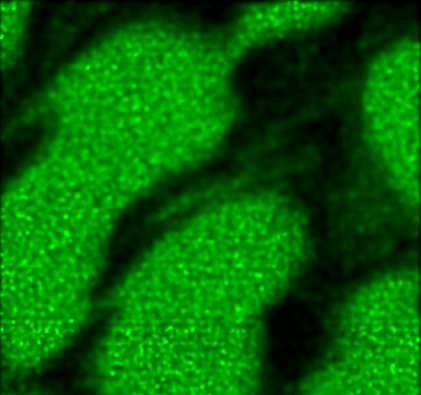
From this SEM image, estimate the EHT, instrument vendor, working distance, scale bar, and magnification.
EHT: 30 kV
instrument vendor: other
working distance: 25.1 mm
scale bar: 20000 nm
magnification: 1.85 K X